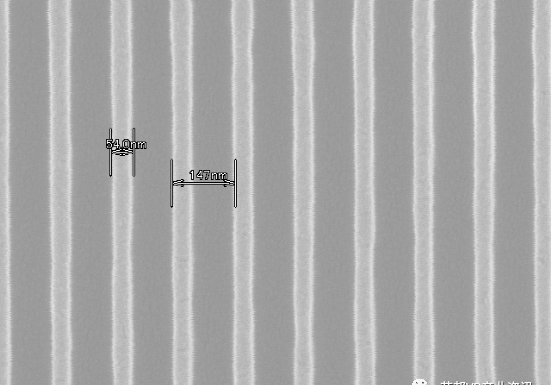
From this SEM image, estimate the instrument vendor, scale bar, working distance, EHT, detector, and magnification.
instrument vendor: Hitachi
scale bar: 500 nm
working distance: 15.3 mm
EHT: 10 kV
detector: SE(U)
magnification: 100 K X